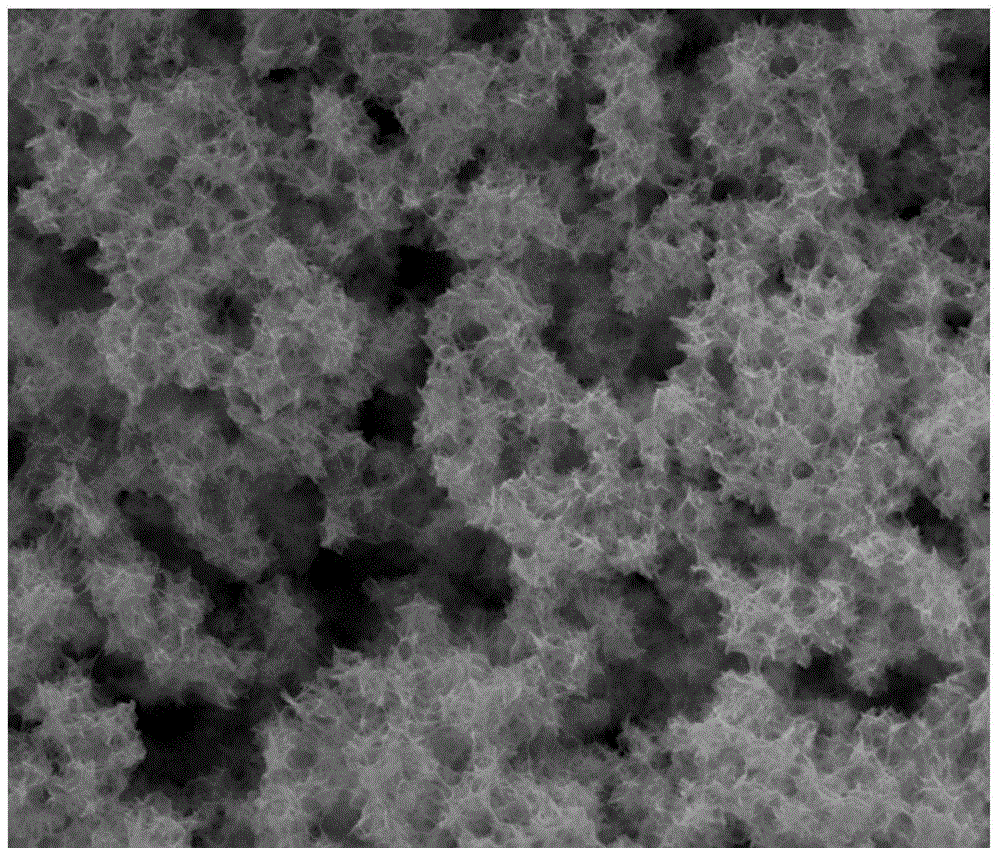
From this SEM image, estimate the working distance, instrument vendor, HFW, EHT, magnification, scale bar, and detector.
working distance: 10.2 mm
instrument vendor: FEI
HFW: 29.8 µm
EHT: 20 kV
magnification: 10 K X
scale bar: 10000 nm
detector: ETD